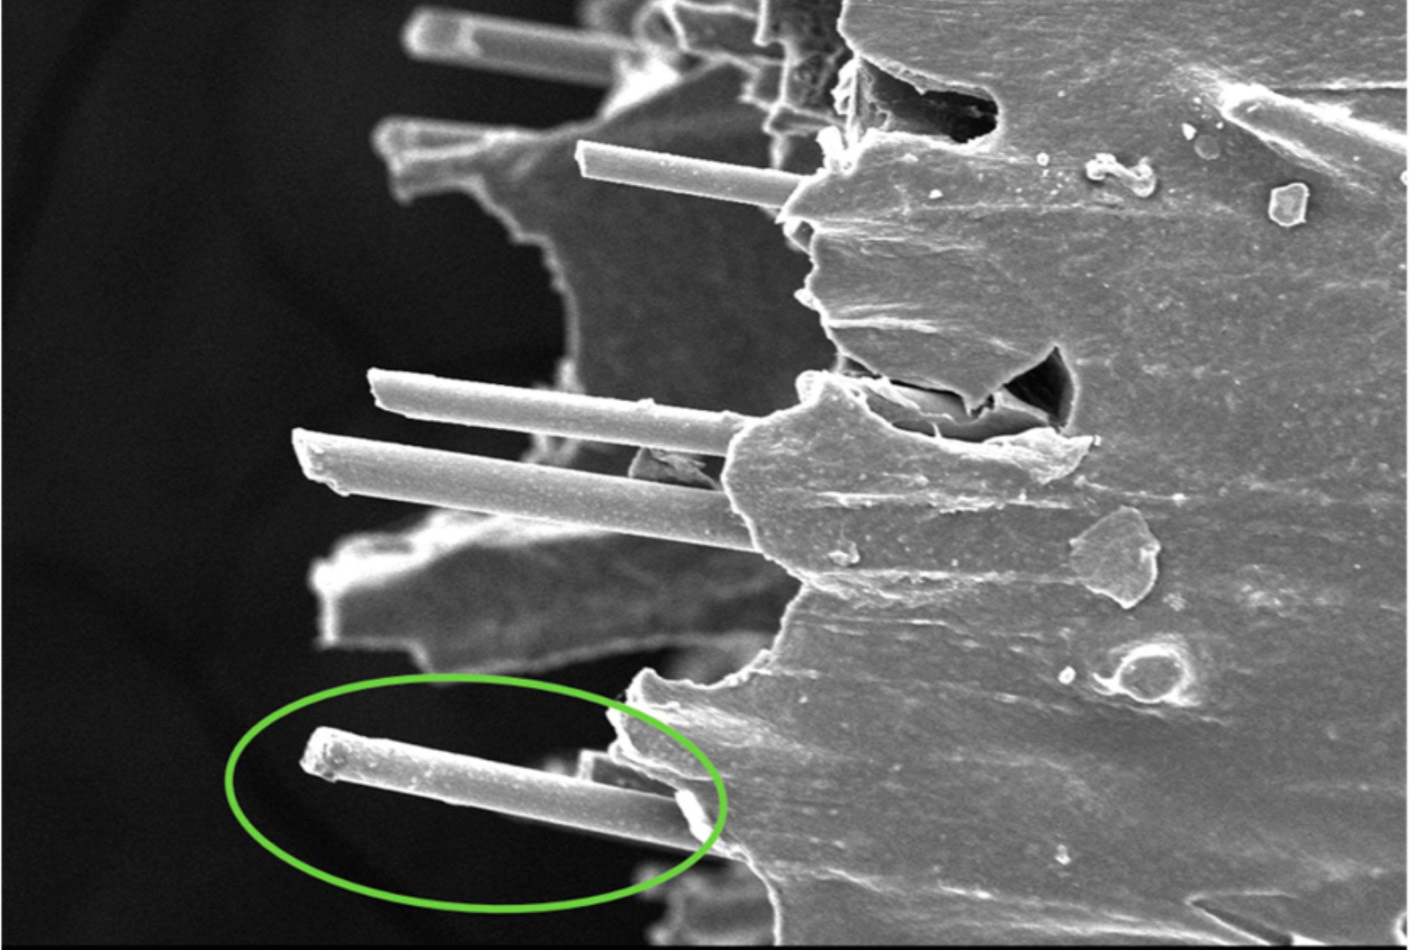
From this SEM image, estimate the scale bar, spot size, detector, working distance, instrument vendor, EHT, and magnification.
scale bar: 100000 nm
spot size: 9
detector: SE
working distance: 11.5 mm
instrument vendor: other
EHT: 12 kV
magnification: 0.5 K X